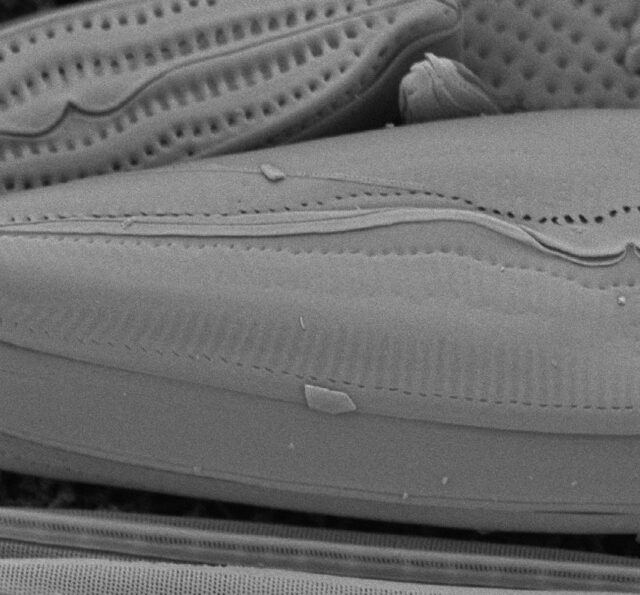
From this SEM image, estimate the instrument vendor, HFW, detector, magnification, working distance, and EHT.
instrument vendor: Thermo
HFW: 41.6 µm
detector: BSD Full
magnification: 12.5 K X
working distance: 10.757 mm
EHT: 10 kV